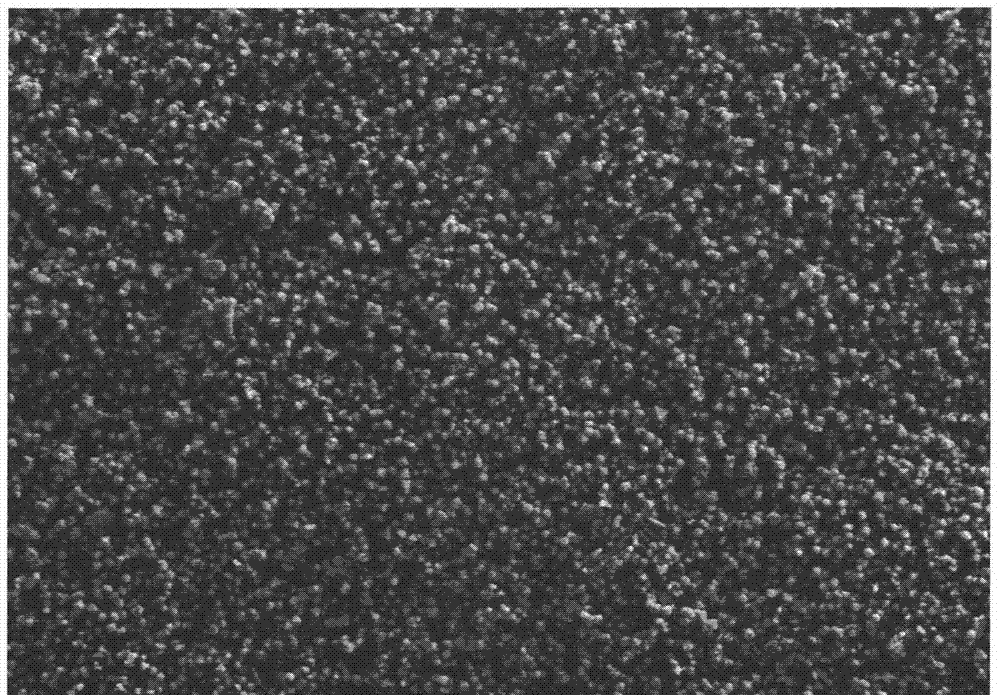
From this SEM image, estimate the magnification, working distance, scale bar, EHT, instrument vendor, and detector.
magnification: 5 K X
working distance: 6.5 mm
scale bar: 1000 nm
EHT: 5 kV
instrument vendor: Zeiss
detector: SE2